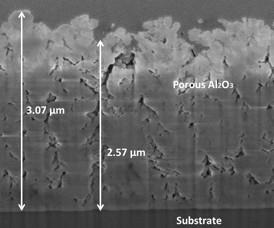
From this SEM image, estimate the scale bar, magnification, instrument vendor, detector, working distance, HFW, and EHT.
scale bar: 500 nm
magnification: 50 K X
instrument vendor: FEI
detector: TLD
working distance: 4.3 mm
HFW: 2.56 µm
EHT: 2 kV